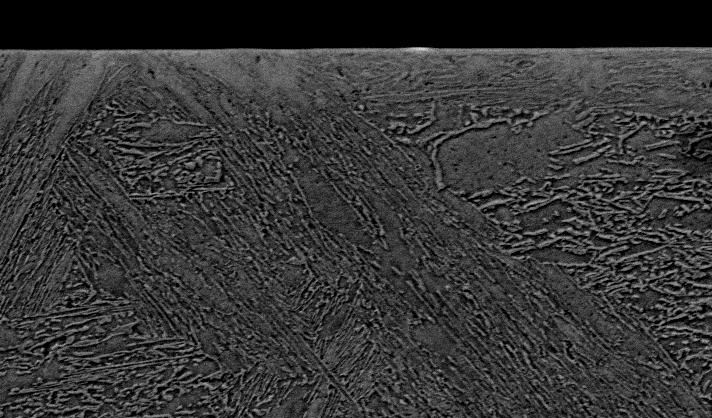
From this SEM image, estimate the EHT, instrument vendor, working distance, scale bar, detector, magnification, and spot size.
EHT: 20 kV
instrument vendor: FEI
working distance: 10.7 mm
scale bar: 20000 nm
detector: BSE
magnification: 1.5 K X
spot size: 5